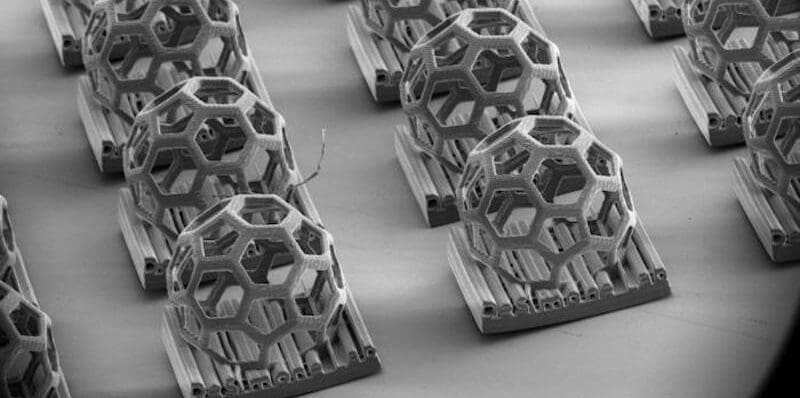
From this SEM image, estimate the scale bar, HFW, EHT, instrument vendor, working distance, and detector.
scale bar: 1000000 nm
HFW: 3190 µm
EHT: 2 kV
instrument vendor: Thermo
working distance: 15.8 mm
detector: ETD